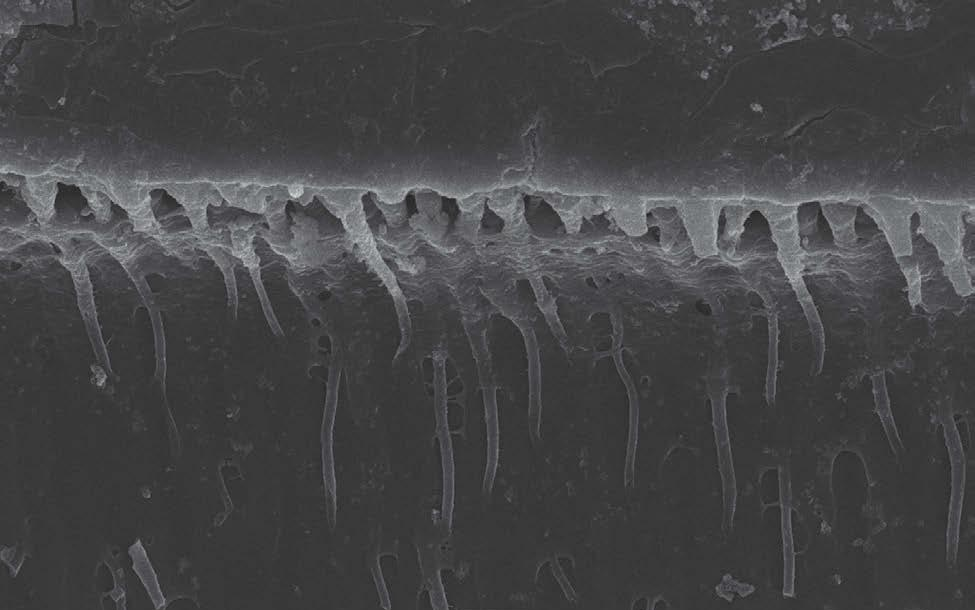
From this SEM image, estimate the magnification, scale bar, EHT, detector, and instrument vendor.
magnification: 1 K X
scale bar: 10000 nm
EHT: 25 kV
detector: SEI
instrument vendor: JEOL